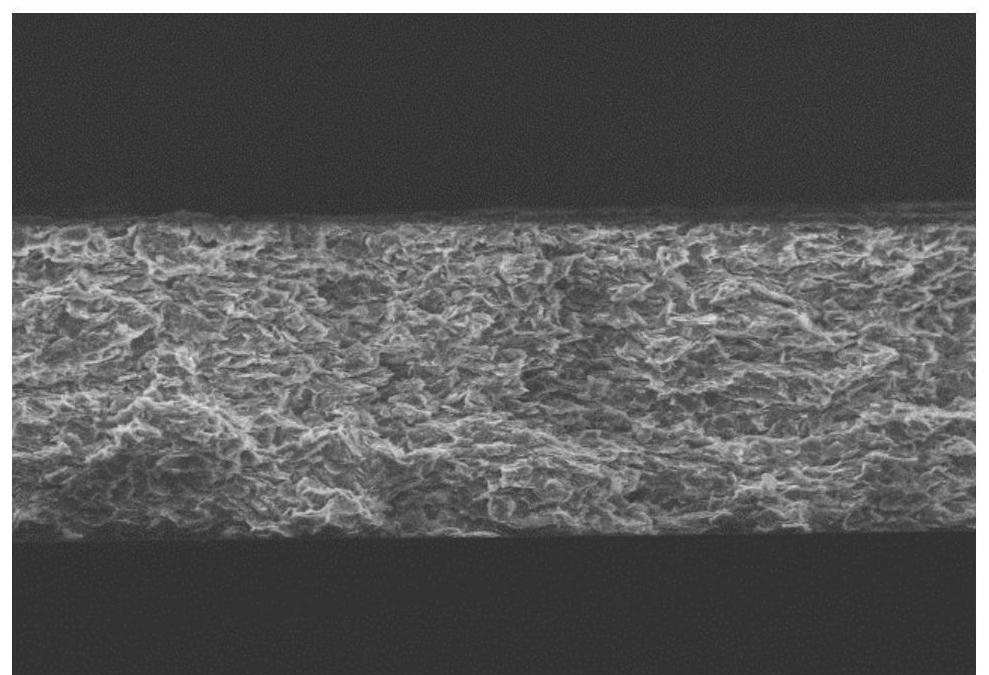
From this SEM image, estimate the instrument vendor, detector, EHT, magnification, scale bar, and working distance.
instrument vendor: Hitachi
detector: SE(M)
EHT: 5 kV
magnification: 2 K X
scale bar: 20000 nm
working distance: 4.7 mm